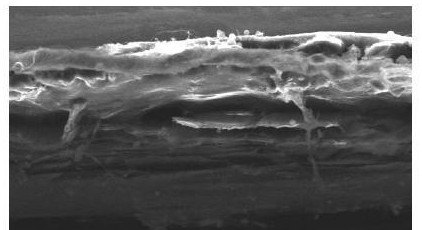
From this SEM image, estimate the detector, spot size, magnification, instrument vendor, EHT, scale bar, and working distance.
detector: SEI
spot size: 55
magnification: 0.25 K X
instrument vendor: JEOL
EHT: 25 kV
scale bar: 100000 nm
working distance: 12 mm